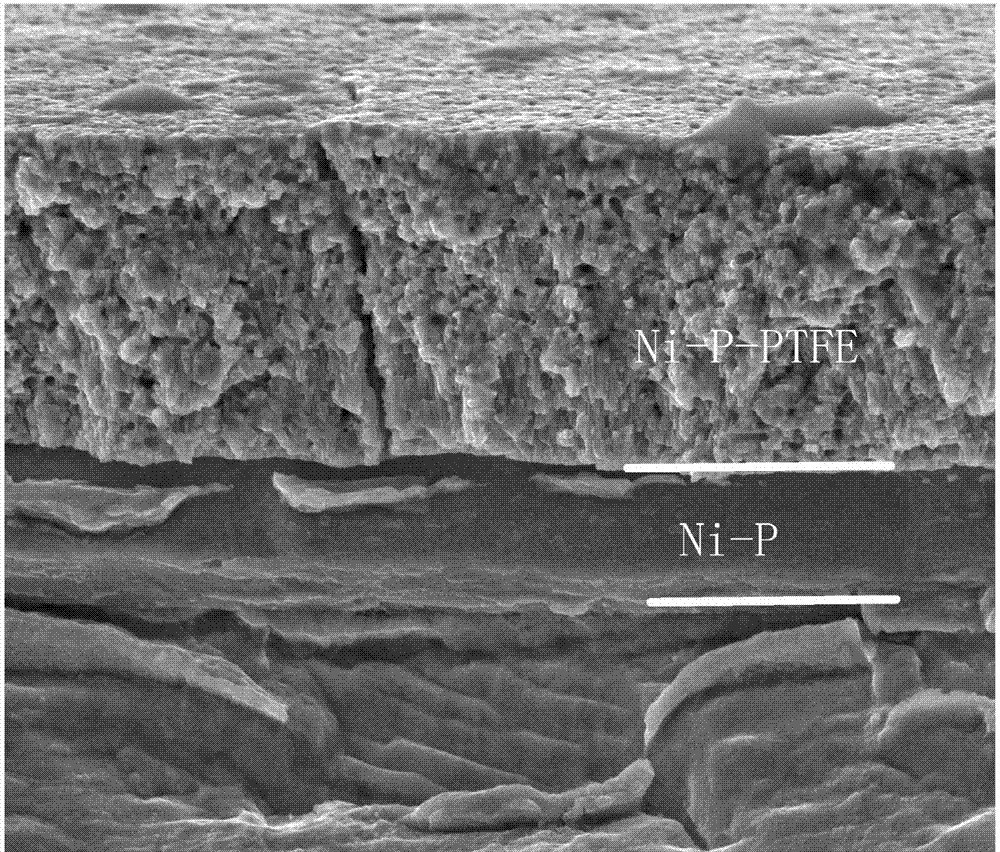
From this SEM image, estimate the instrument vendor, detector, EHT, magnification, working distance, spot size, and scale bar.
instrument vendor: FEI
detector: ETD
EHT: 10 kV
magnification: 25 K X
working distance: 8 mm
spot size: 3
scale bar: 5000 nm